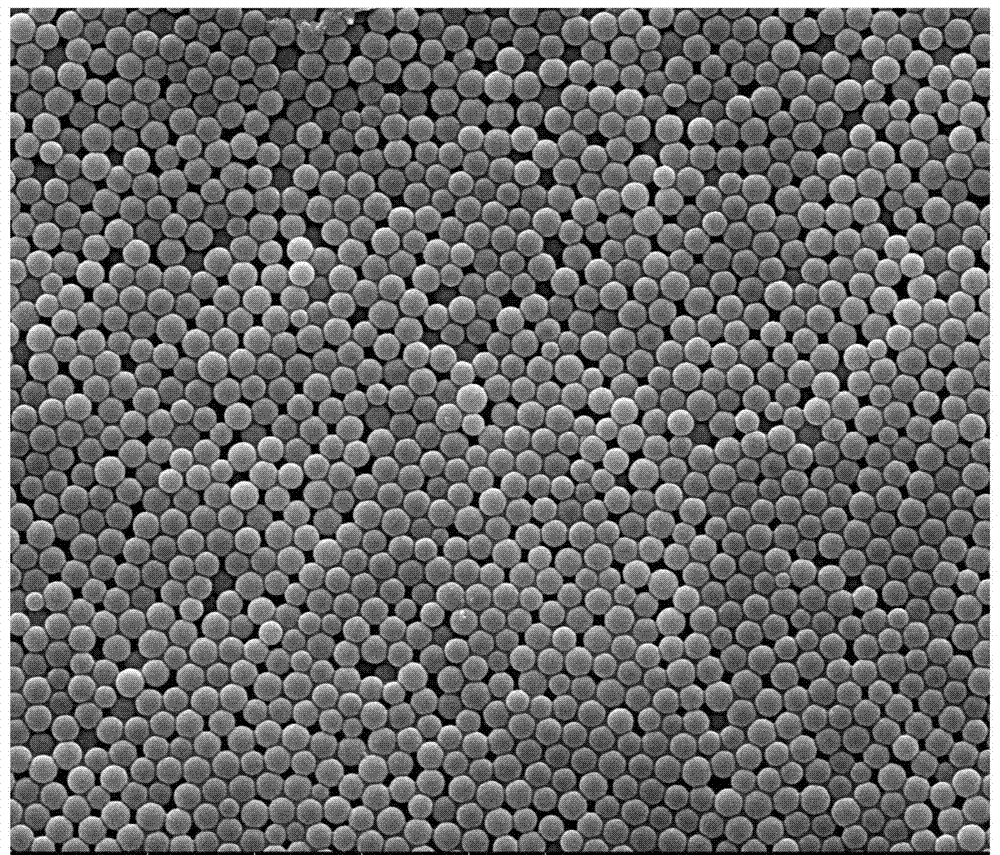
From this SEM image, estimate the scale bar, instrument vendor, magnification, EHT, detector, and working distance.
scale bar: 5000 nm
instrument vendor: FEI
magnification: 20 K X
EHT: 10 kV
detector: SE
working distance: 11.2 mm